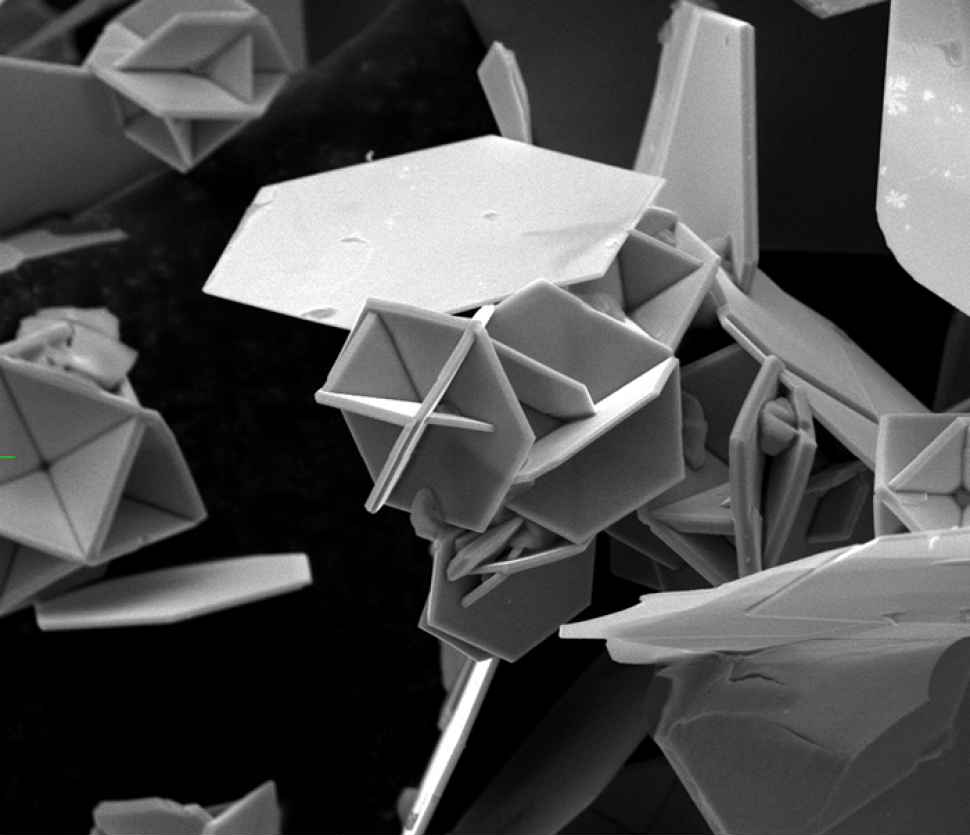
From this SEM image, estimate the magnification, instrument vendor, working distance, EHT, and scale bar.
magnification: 6 K X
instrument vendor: FEI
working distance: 8.7 mm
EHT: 10 kV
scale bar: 20000 nm